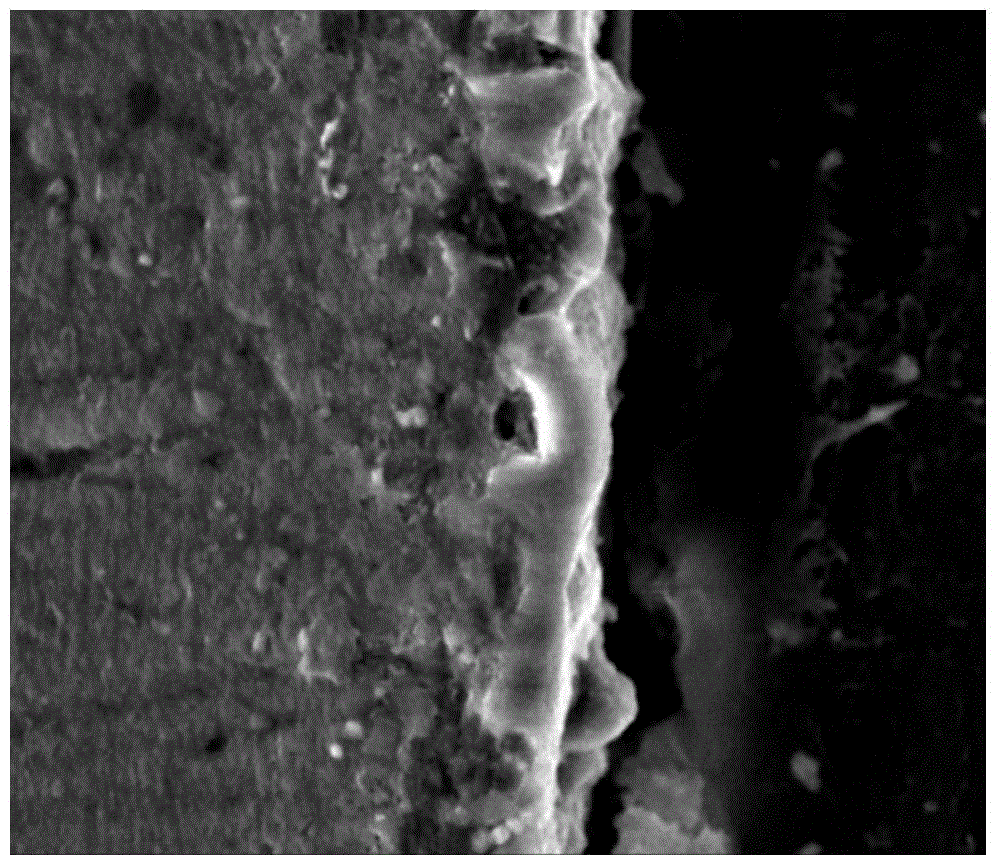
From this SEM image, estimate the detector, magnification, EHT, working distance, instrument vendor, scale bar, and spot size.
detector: ETD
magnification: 8 K X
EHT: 15 kV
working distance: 10.3 mm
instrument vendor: FEI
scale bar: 10000 nm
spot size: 4.5